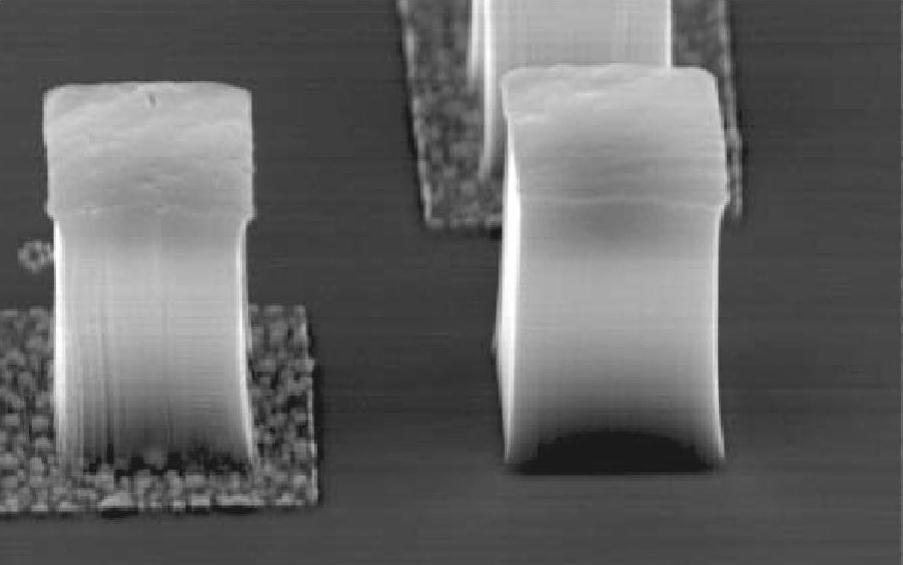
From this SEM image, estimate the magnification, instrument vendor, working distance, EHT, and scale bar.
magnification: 1.6 K X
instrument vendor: JEOL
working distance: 11 mm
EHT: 15 kV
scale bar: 10000 nm